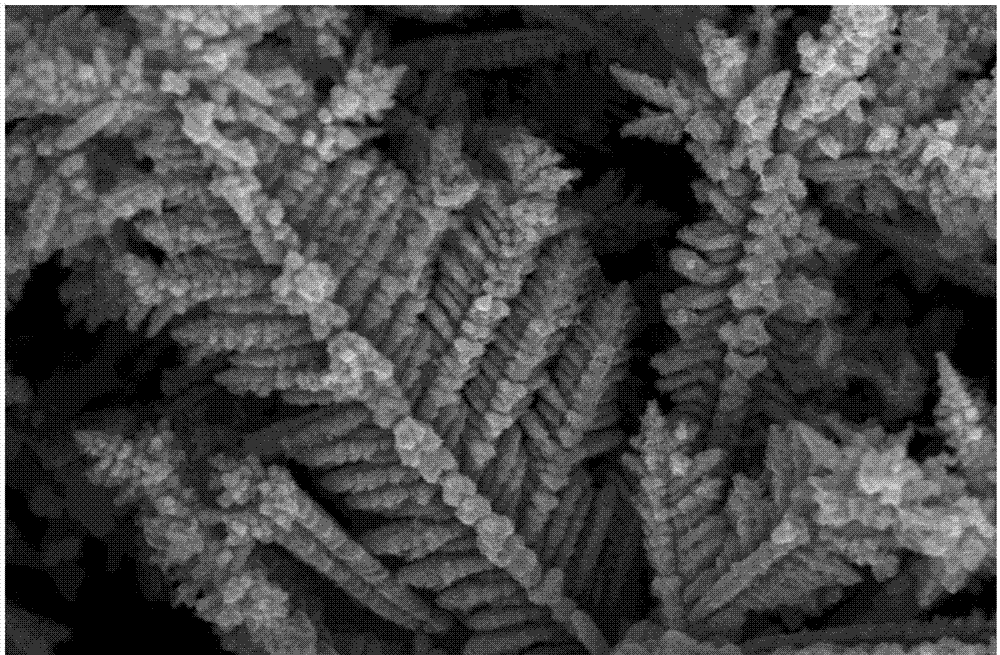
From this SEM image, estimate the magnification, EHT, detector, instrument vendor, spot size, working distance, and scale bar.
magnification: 50 K X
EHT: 10 kV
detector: TLD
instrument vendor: FEI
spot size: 3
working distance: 4.9 mm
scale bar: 3000 nm